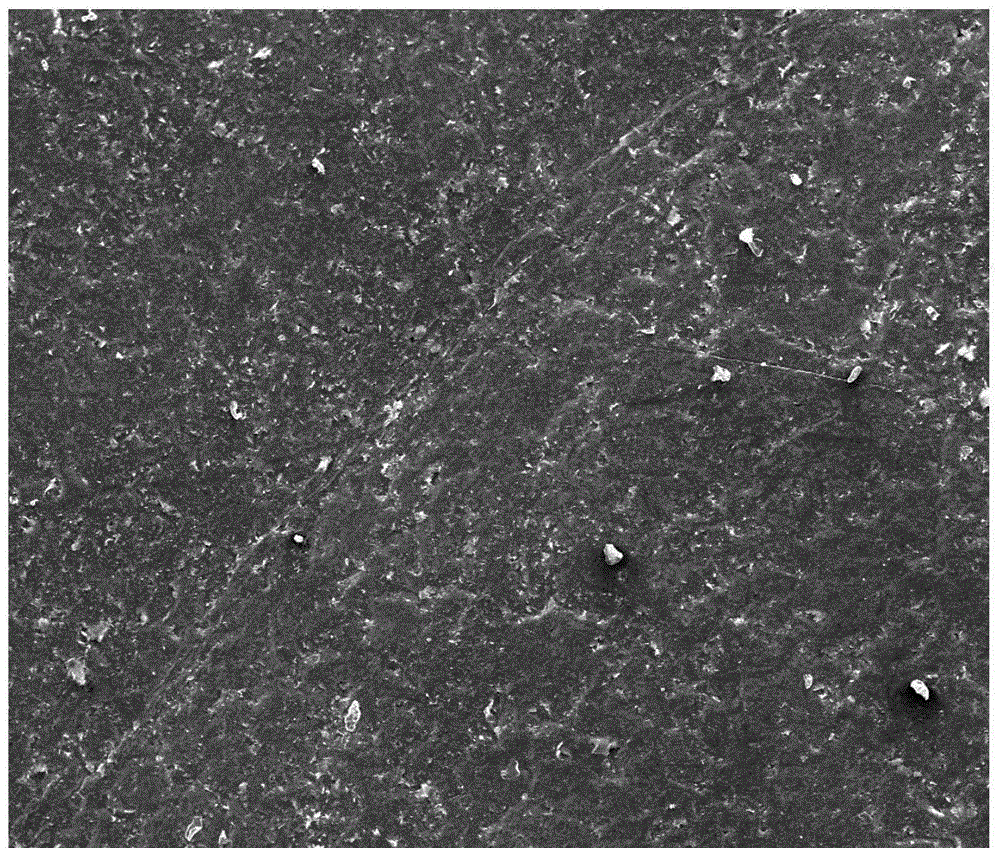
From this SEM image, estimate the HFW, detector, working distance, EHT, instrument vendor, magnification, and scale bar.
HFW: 1270 µm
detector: ETD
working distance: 11.4 mm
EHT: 10 kV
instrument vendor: FEI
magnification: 0.1 K X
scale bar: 500000 nm